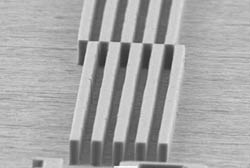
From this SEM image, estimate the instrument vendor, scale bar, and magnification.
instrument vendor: JEOL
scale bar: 20000 nm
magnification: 0.6 K X